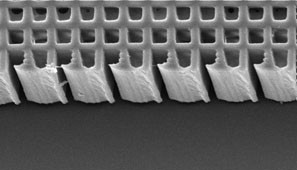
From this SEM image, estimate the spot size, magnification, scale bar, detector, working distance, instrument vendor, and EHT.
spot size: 5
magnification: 1 K X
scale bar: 50000 nm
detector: SE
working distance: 10.8 mm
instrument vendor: FEI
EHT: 20 kV